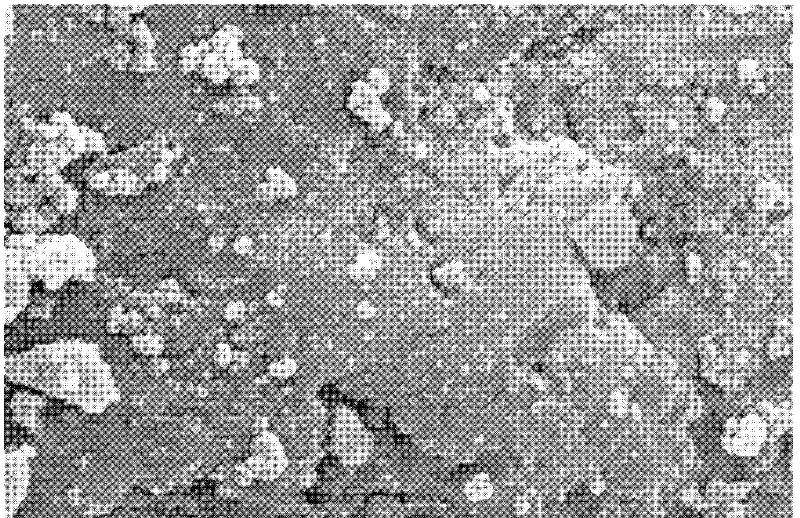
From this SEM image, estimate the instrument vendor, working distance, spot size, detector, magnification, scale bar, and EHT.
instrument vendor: FEI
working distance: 10.2 mm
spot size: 4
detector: SE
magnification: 20 K X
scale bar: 2000 nm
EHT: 25 kV